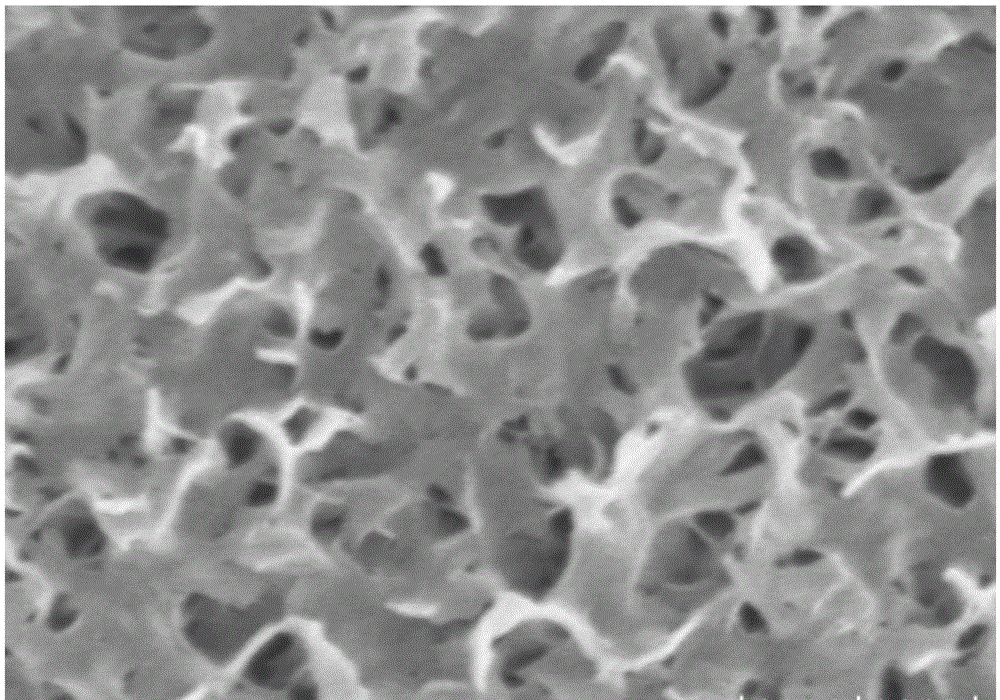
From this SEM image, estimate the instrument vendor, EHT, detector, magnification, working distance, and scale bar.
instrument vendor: Hitachi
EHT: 5 kV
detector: SE(UL)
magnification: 60 K X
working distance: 10.1 mm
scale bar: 500 nm